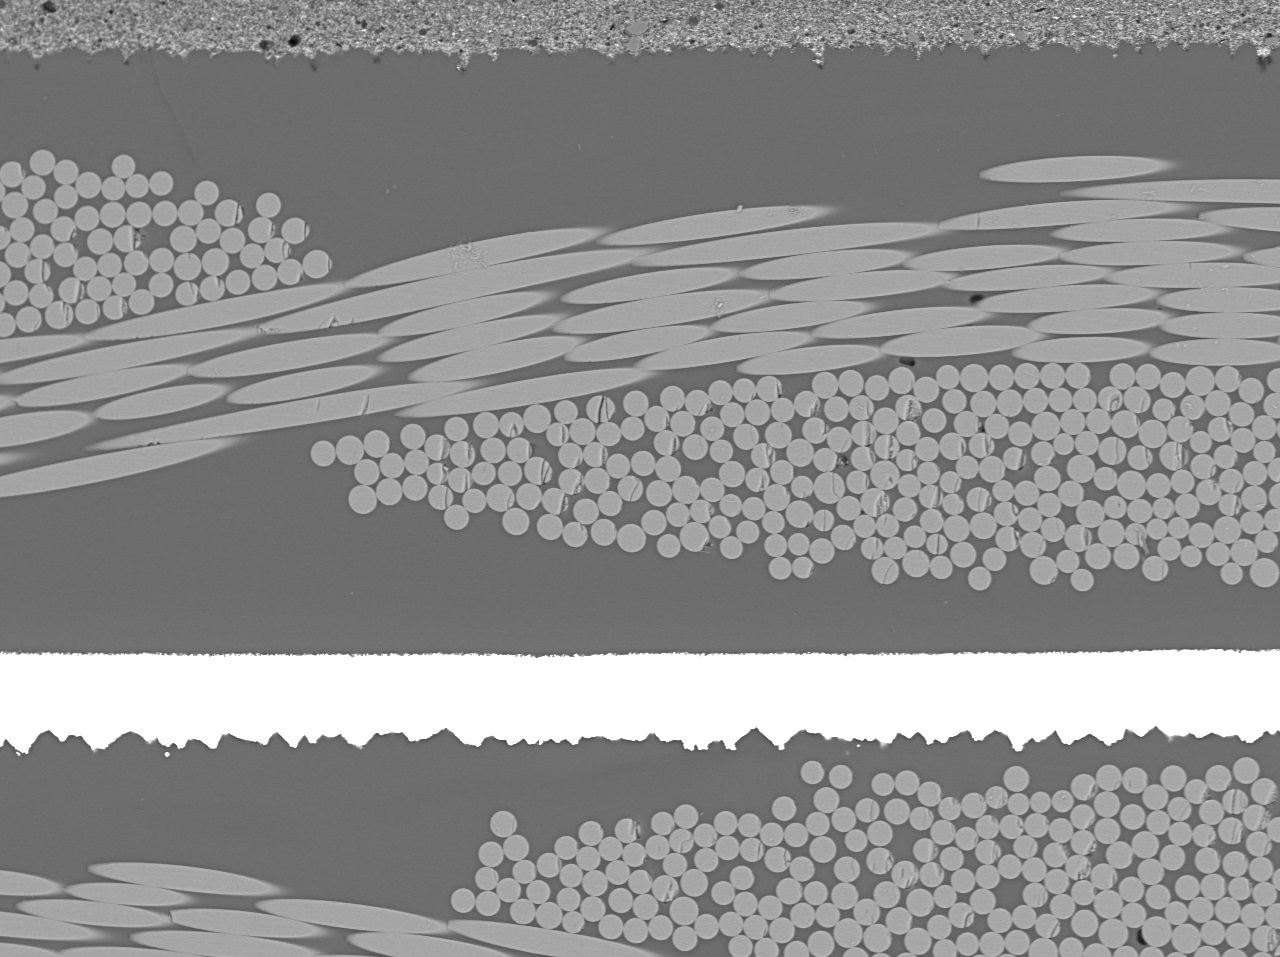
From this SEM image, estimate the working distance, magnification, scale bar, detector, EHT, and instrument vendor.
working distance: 12.9 mm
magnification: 0.27 K X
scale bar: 50000 nm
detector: BED-C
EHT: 15 kV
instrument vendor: JEOL